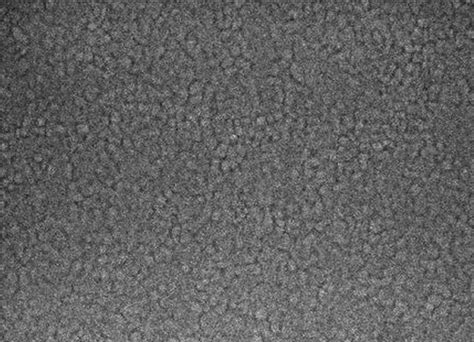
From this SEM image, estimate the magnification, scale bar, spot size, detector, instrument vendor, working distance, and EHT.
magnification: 80 K X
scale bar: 200 nm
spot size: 2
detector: TLD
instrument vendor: FEI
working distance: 3.3 mm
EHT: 10 kV